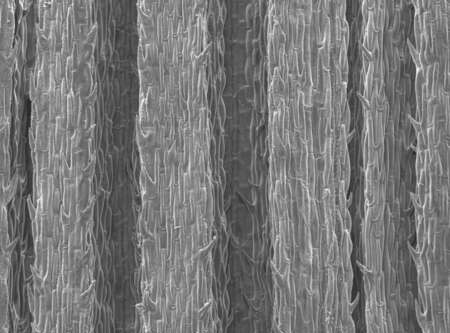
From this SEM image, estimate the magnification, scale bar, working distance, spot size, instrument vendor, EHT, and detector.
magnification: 0.1 K X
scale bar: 200000 nm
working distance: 9.7 mm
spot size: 3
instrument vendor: FEI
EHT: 10 kV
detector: SE